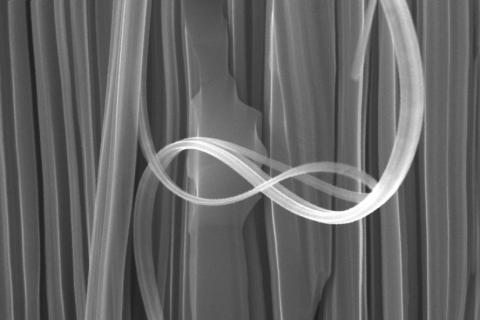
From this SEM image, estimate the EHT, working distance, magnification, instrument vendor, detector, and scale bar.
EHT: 15 kV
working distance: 4.2 mm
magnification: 30 K X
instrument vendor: Zeiss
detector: InLens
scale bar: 200 nm